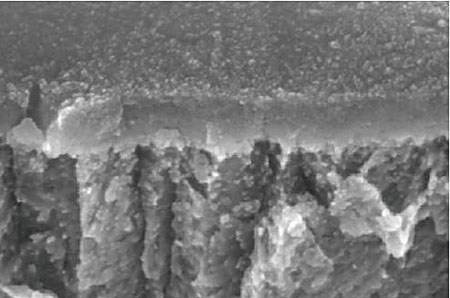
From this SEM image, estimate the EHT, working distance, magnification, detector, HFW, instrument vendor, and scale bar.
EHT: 10 kV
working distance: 6.4 mm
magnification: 50 K X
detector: InLens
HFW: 2.287 µm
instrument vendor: Zeiss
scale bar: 100 nm